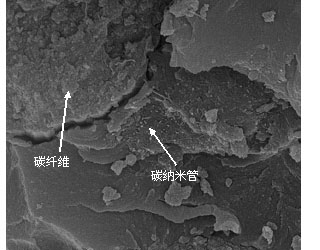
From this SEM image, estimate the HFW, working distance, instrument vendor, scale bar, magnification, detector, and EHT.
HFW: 6.76 µm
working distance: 11.9 mm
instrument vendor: FEI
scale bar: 1000 nm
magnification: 40 K X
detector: SE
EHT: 20 kV